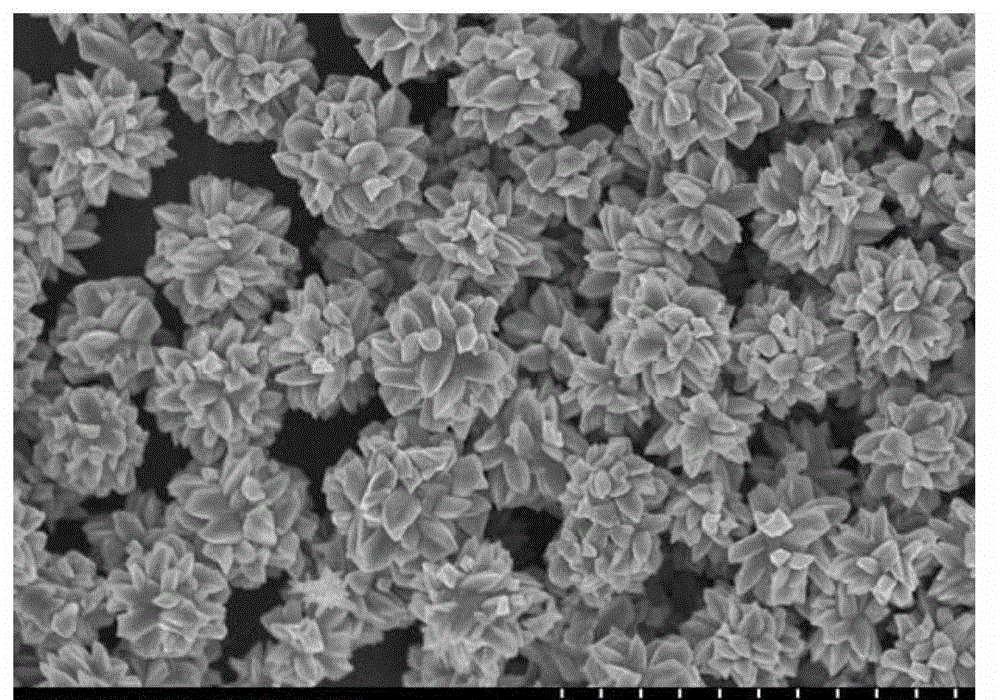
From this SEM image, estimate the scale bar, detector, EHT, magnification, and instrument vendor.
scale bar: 4000 nm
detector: SE(U)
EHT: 5 kV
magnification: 13 K X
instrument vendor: Hitachi